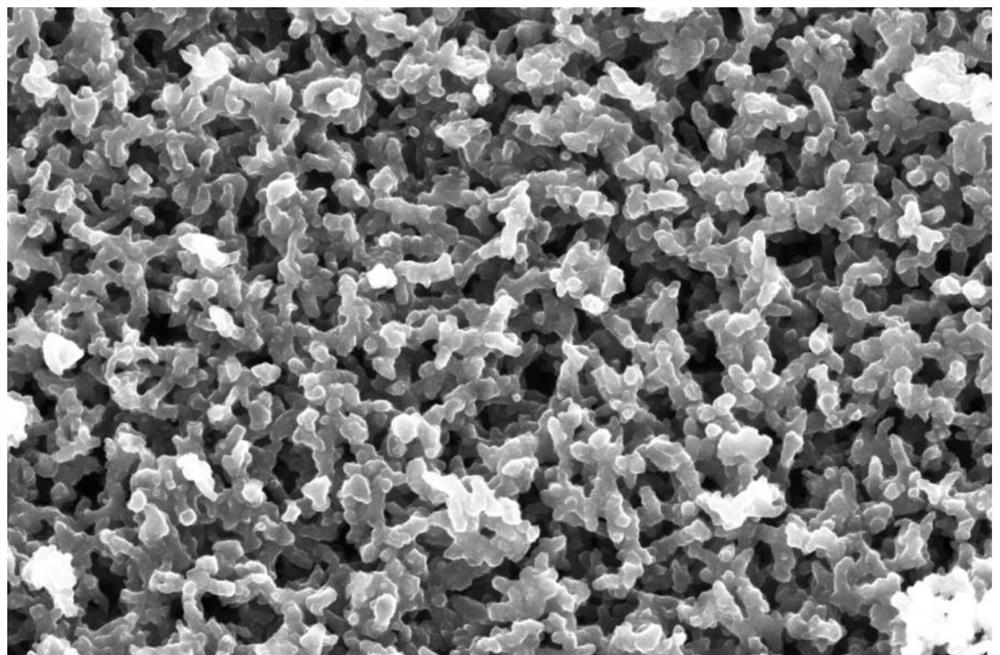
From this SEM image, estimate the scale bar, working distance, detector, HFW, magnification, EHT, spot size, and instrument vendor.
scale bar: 1000 nm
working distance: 10.3 mm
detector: T2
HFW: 6.91 µm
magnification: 60 K X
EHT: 5 kV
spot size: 6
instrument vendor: Thermo